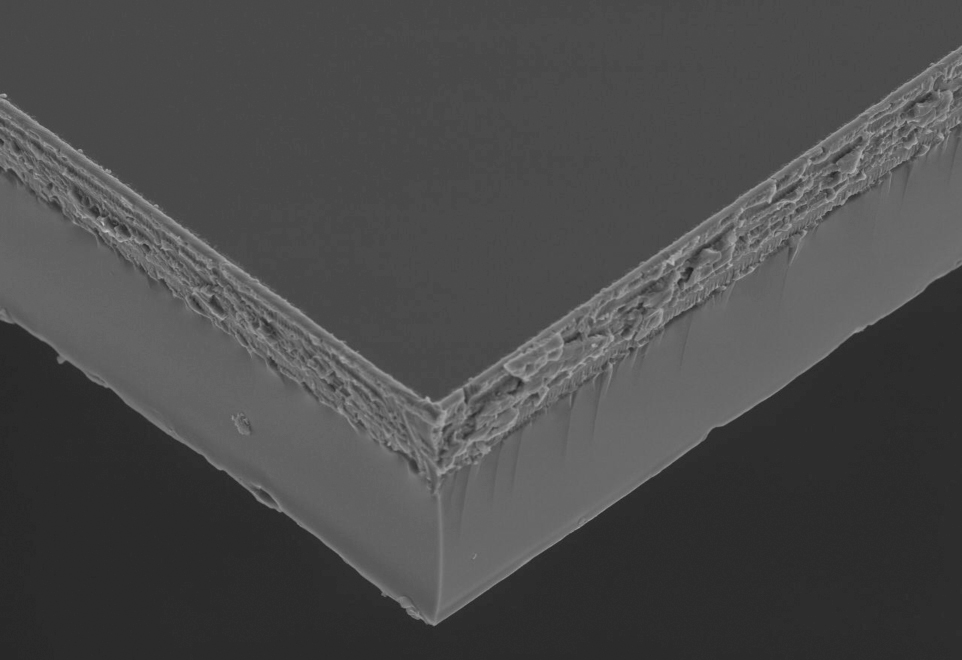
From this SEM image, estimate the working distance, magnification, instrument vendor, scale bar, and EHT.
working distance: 28.1 mm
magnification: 0.5 K X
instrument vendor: JEOL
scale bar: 20000 nm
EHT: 20 kV